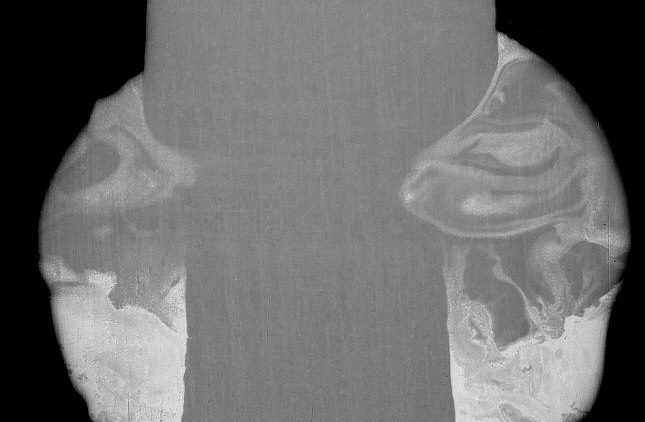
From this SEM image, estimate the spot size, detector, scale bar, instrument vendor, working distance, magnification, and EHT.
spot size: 4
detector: BSE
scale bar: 200000 nm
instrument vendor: FEI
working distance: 10.4 mm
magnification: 0.14 K X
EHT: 20 kV